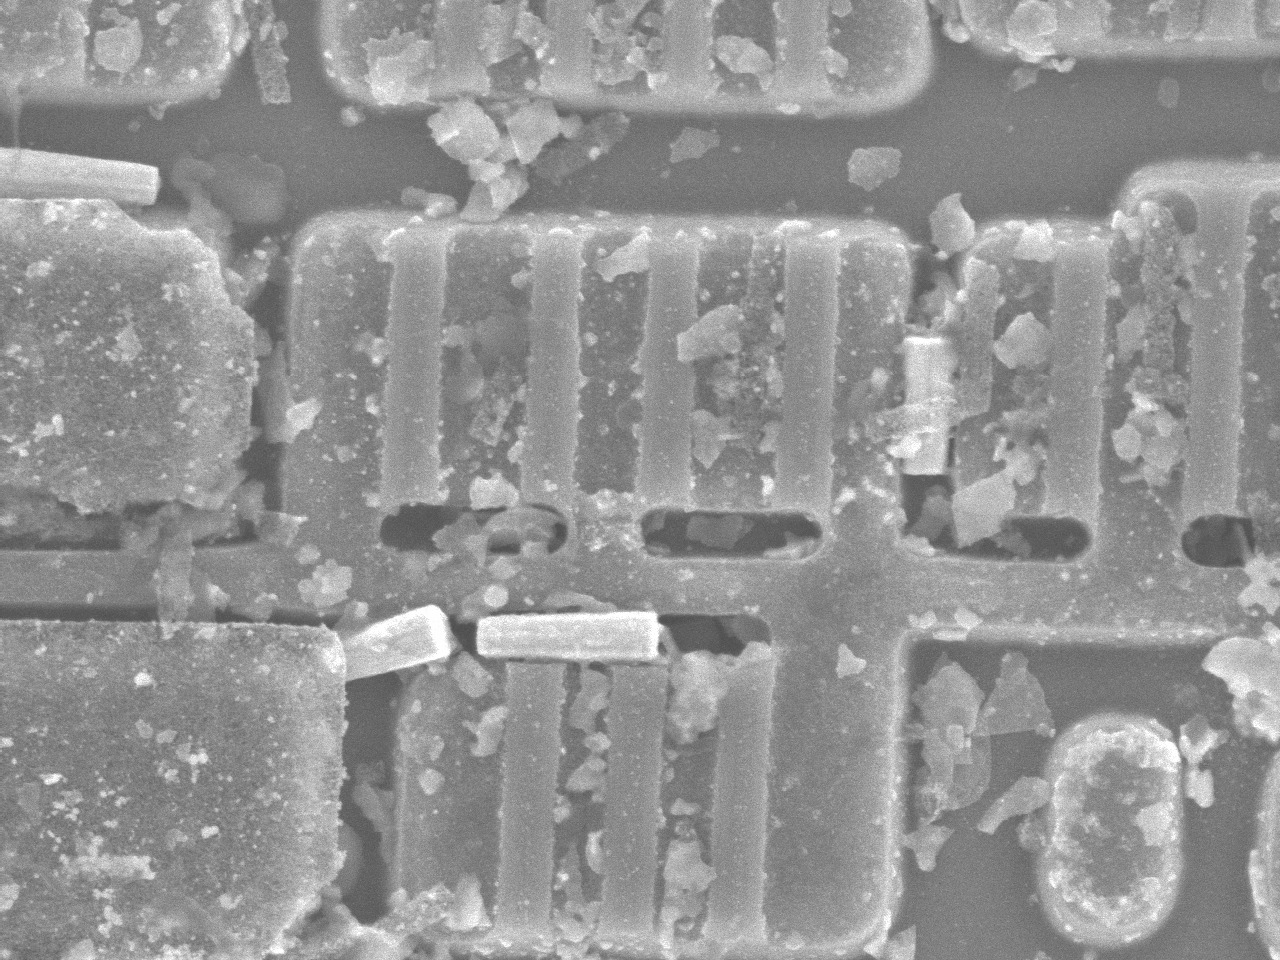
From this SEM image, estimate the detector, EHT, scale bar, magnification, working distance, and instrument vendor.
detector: SEI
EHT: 5 kV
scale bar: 1000 nm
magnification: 18 K X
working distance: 15.3 mm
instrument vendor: JEOL